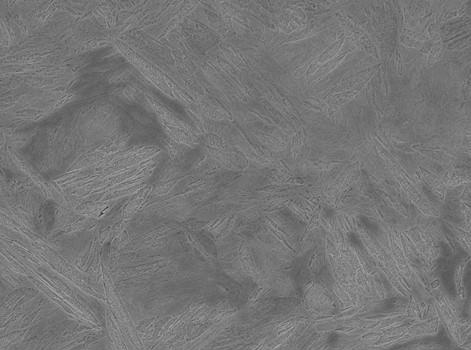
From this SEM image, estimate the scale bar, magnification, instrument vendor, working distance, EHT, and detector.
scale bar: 10000 nm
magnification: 2.5 K X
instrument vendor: JEOL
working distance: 9.8 mm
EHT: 15 kV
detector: SEI